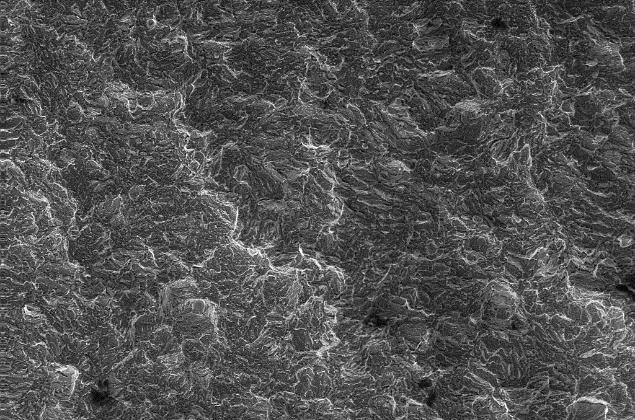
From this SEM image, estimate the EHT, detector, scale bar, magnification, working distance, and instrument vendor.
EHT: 20 kV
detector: SE1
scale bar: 200000 nm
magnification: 0.2 K X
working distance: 21 mm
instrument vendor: Zeiss